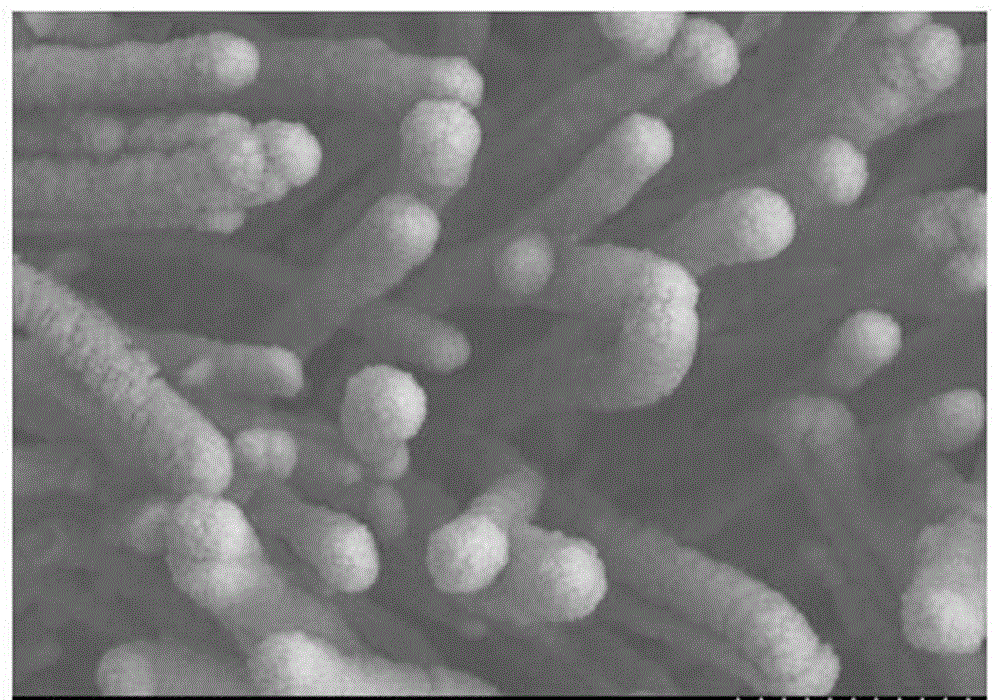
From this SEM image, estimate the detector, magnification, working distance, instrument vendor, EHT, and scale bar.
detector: SE(M)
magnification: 30 K X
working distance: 12.4 mm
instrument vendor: Hitachi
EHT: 5 kV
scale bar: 1000 nm